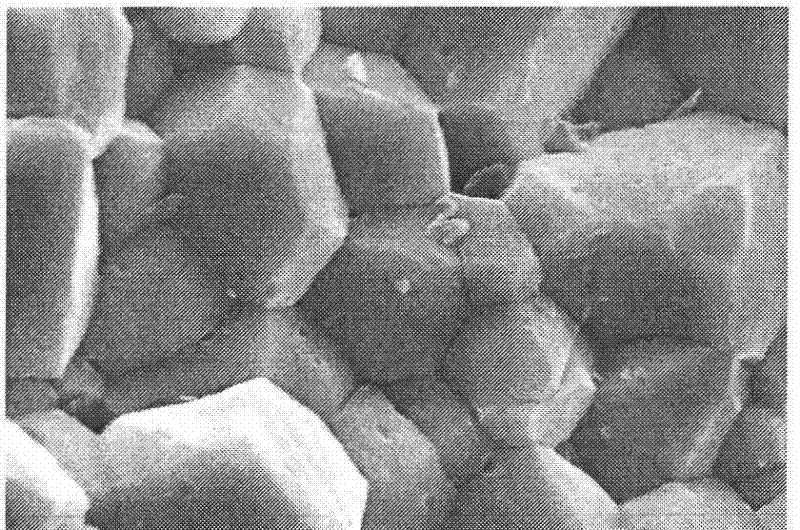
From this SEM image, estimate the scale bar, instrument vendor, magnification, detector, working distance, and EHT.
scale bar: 3000 nm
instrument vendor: Zeiss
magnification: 20 K X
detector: SE1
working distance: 12 mm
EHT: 25 kV